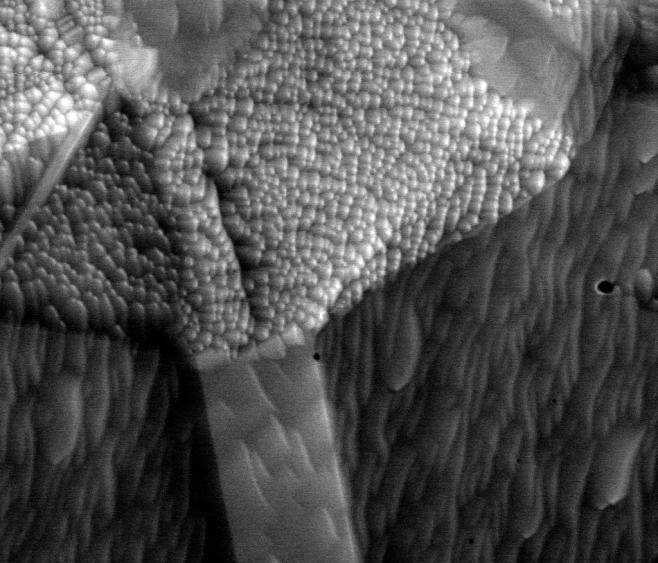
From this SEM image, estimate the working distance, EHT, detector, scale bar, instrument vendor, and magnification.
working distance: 10.1 mm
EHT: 30 kV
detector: ETD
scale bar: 4000 nm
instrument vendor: FEI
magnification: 15 K X